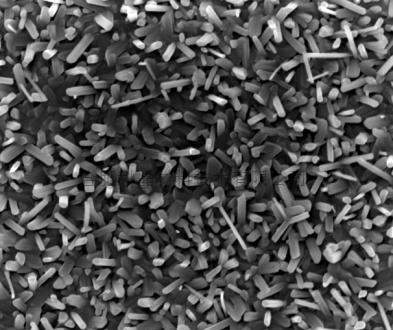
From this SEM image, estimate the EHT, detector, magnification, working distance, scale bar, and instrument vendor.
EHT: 20 kV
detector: TLD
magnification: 20 K X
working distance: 6 mm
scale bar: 2000 nm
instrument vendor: FEI